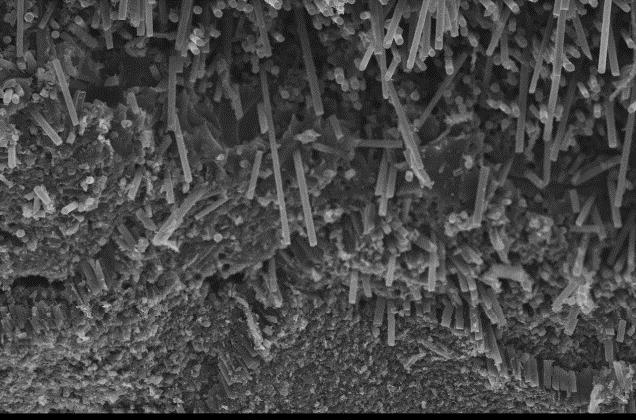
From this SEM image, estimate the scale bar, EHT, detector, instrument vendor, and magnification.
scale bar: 100000 nm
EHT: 30 kV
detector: SEI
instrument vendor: JEOL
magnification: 0.2 K X